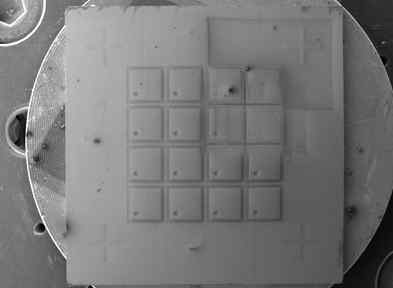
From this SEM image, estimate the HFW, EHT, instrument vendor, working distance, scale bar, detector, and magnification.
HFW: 15800 µm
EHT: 10 kV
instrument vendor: Tescan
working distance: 22.12 mm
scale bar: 5000000 nm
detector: SE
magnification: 0.018 K X